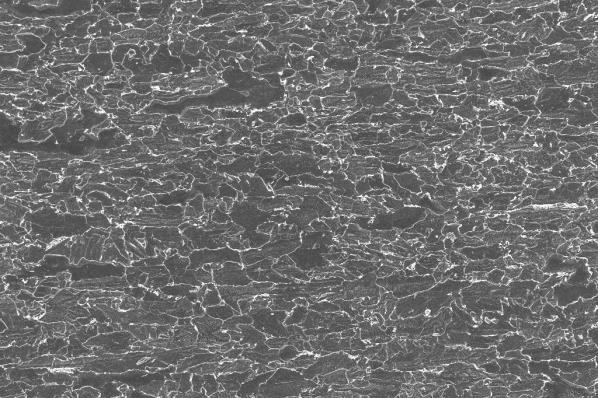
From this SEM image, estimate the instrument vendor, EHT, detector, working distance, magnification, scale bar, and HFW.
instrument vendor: Zeiss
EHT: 5 kV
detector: InLens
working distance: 5.1 mm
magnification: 5 K X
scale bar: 2000 nm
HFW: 59.59 µm